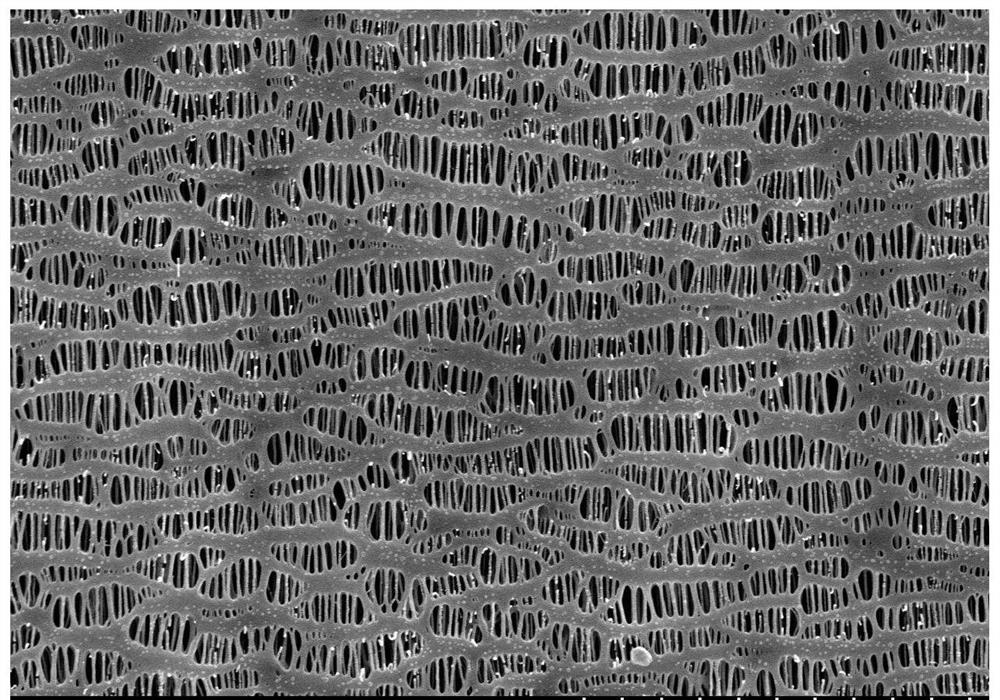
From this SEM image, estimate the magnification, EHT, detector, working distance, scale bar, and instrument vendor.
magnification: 10 K X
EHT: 5 kV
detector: SE(M)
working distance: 8.2 mm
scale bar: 5000 nm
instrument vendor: Hitachi